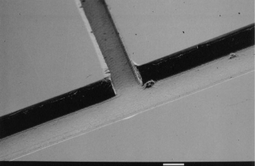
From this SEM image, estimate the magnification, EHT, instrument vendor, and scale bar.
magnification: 0.1 K X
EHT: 10 kV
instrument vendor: JEOL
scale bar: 100000 nm